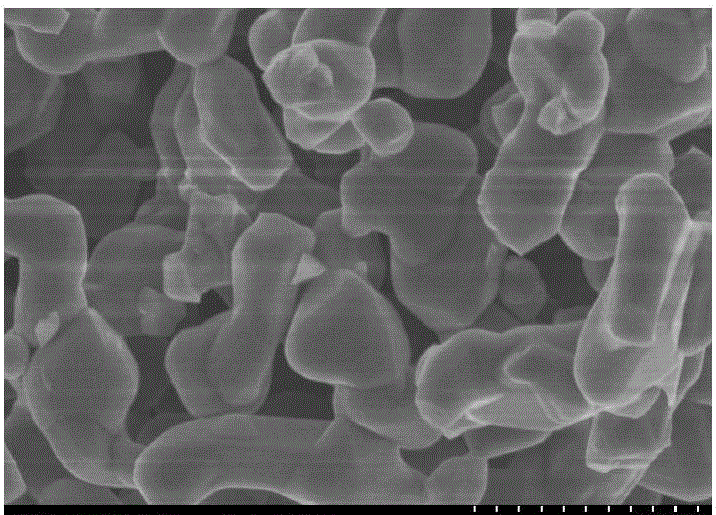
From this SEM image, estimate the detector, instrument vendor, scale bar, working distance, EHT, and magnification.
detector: SE(M)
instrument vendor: Hitachi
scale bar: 1000 nm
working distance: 8 mm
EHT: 3 kV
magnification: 40 K X